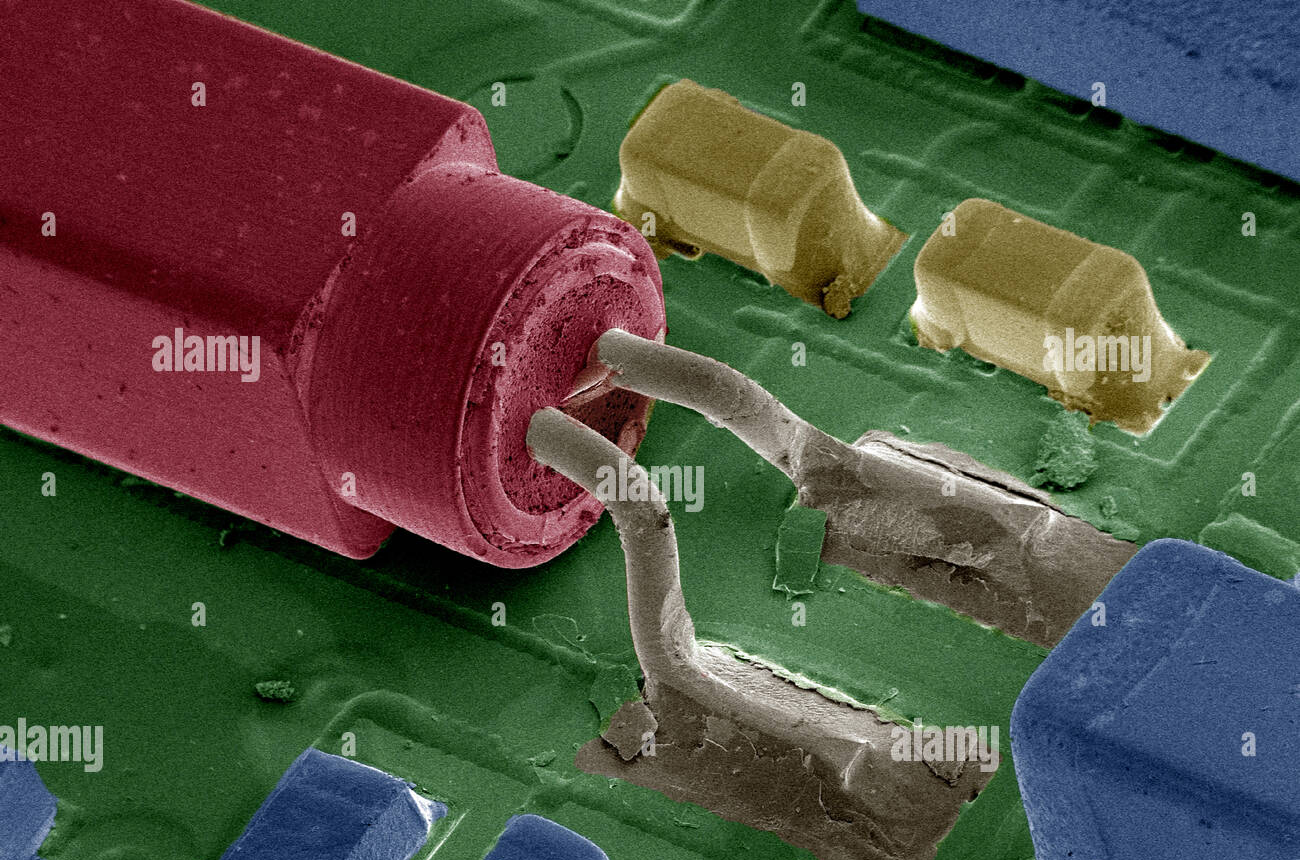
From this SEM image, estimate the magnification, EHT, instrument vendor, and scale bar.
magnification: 0.02 K X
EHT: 10 kV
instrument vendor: JEOL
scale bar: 2000000 nm